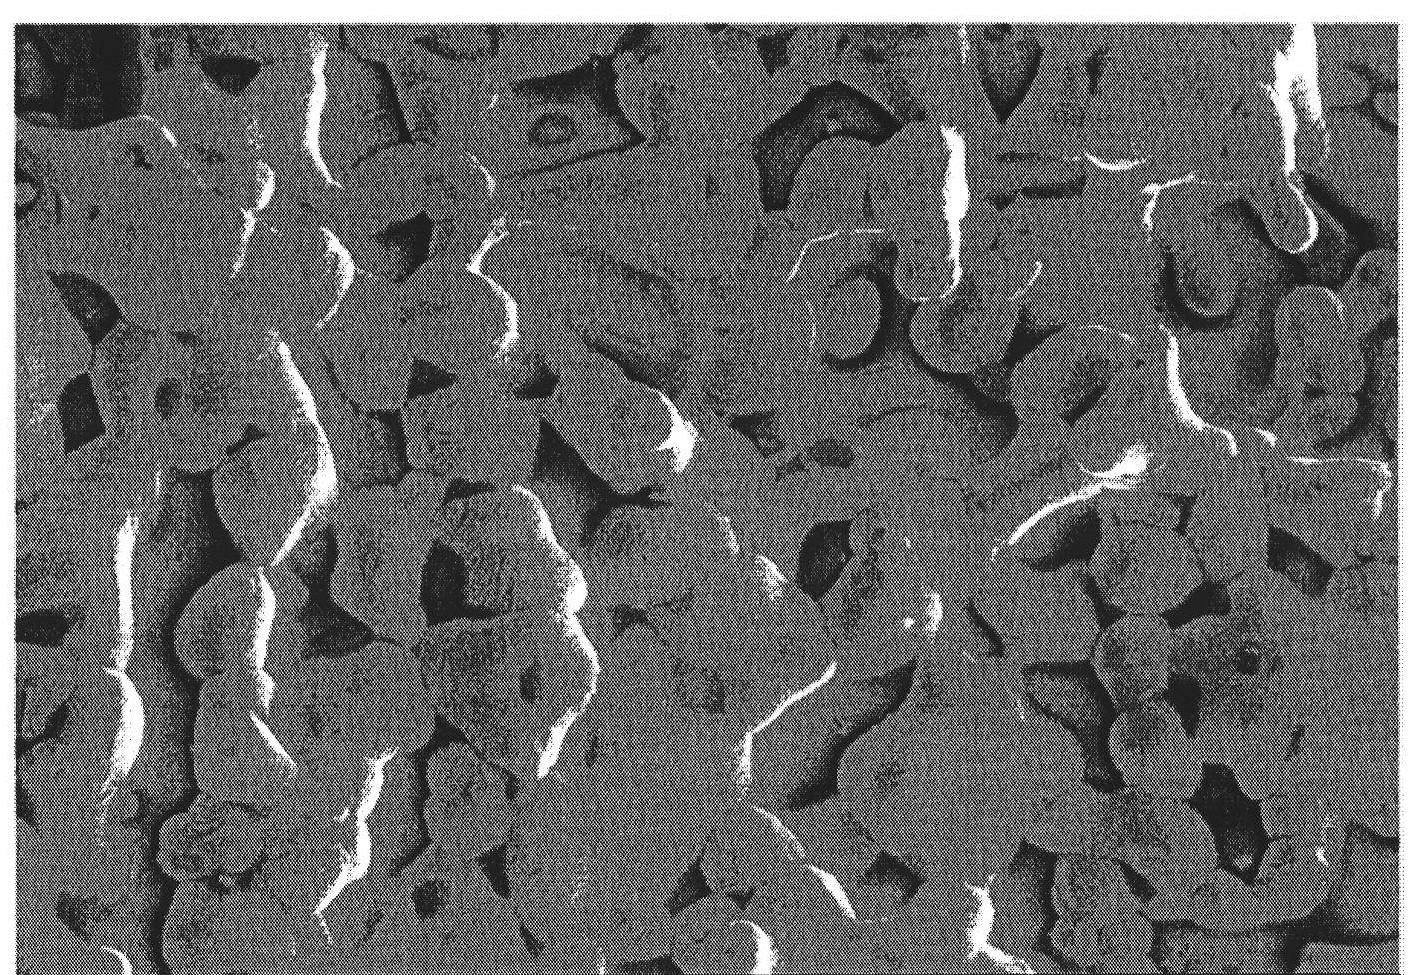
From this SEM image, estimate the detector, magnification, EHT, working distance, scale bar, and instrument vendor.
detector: SE(M)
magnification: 5 K X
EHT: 5 kV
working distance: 7.9 mm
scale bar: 10000 nm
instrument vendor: Hitachi